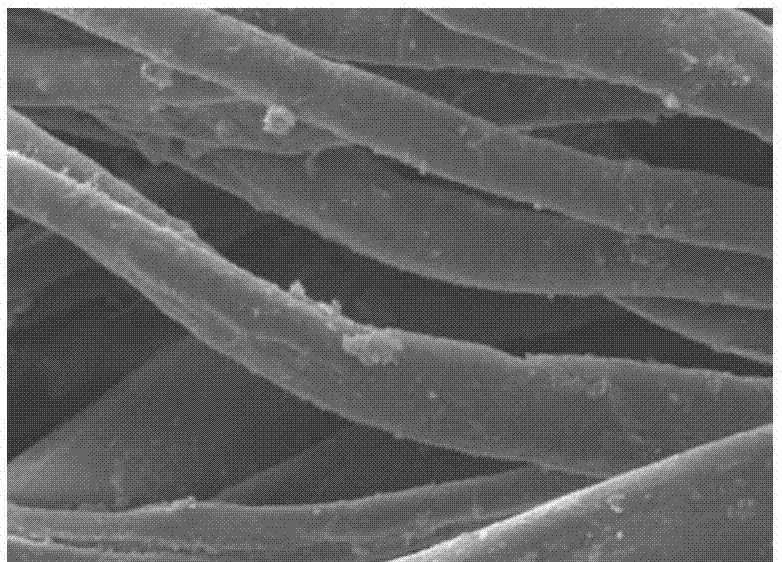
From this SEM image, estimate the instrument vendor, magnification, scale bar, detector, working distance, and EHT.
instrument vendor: JEOL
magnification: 1.3 K X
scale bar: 10000 nm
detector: SEI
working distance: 8 mm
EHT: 10 kV